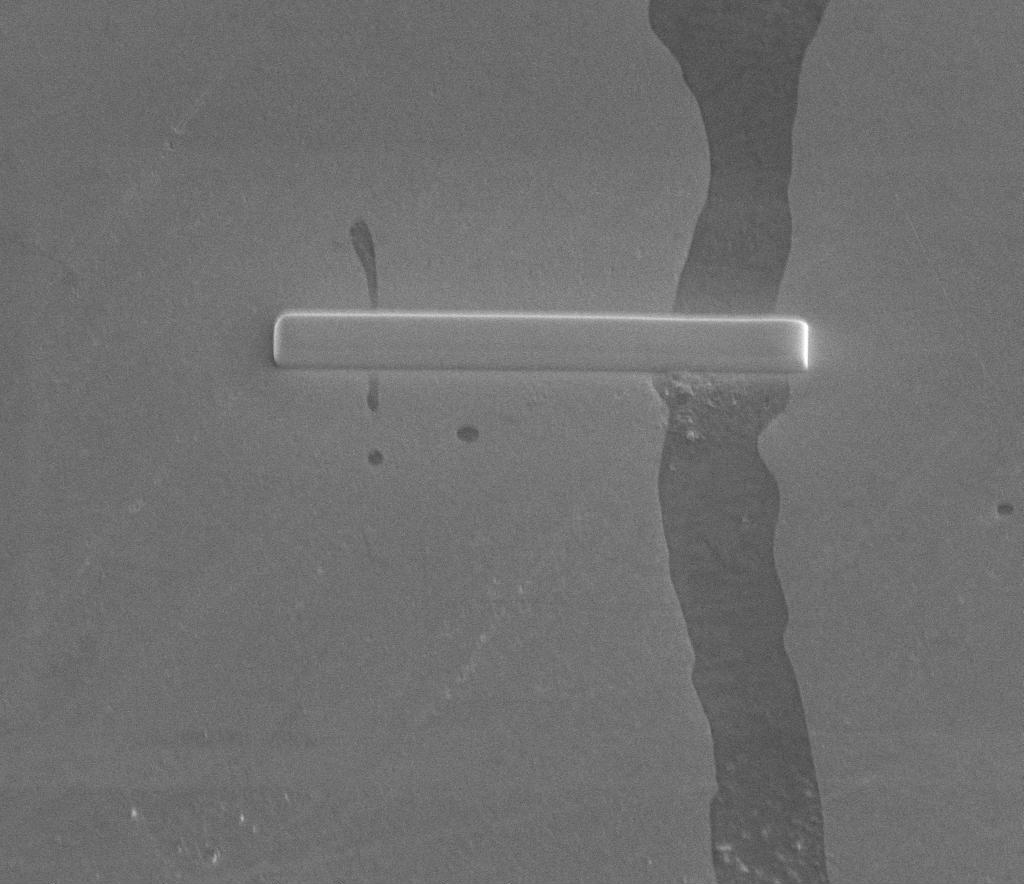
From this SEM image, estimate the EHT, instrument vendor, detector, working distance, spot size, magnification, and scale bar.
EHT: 10 kV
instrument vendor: FEI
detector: ETD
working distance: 10 mm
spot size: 4.5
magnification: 2.5 K X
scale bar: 20000 nm